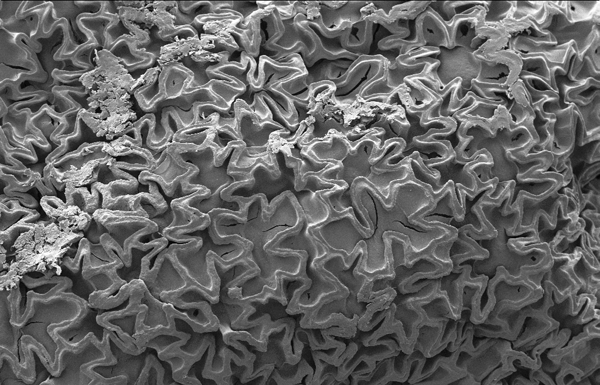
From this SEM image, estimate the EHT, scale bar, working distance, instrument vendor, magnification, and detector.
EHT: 5 kV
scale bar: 10000 nm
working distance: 20 mm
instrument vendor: Zeiss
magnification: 1.46 K X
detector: SE2